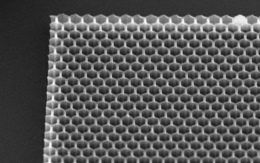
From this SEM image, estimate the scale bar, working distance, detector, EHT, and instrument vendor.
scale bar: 20000 nm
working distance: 6.5 mm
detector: SE1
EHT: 15.01 kV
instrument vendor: Zeiss